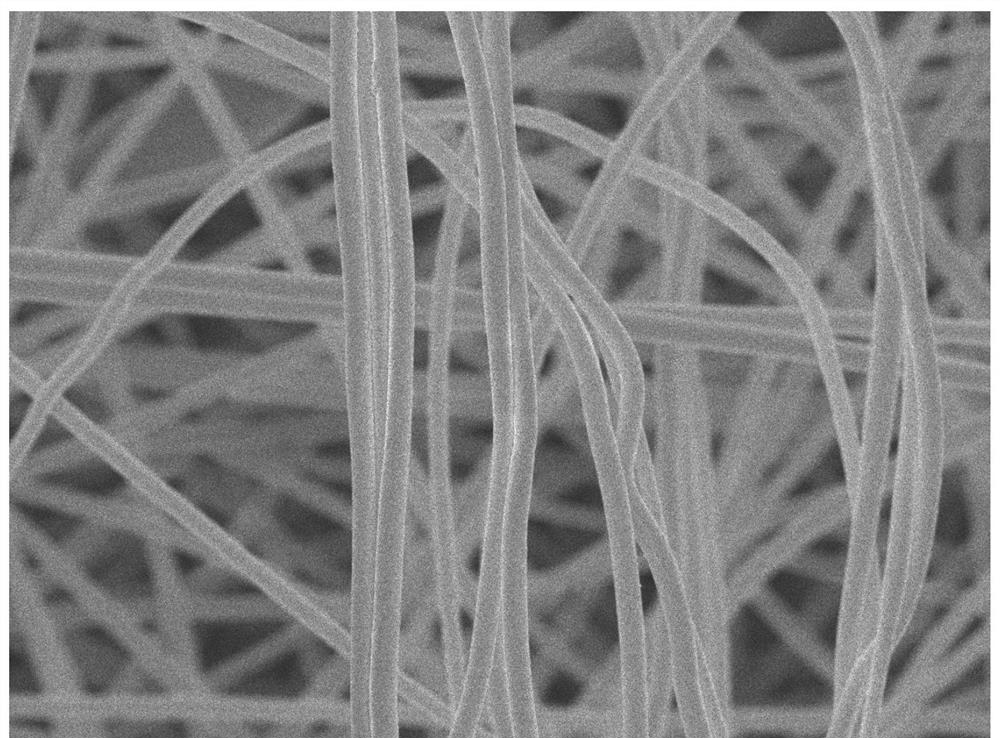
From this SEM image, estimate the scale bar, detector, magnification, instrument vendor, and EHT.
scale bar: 1000 nm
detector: SEI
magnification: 10 K X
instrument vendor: JEOL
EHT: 5 kV